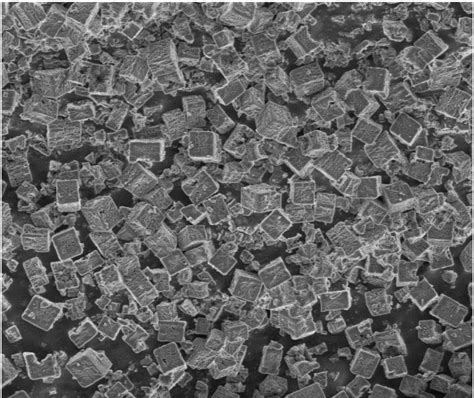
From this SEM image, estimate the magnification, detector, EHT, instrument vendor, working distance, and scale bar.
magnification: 5 K X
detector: SE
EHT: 3 kV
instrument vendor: FEI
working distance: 4.7 mm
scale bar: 10000 nm